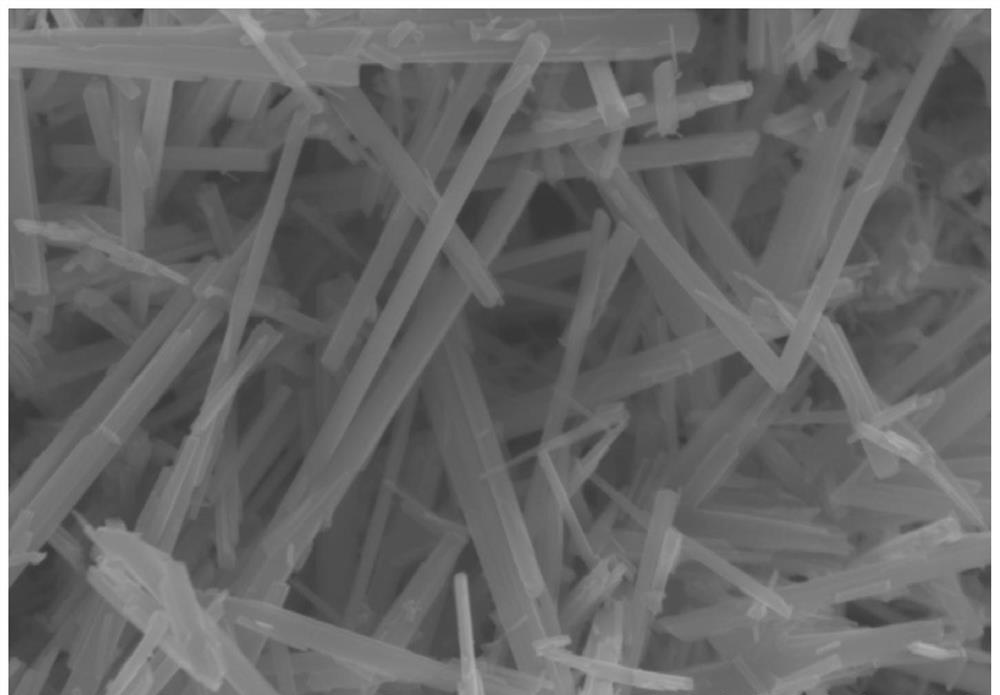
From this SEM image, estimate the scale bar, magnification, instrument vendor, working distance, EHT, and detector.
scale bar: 1000 nm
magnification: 45 K X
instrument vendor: Hitachi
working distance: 9.6 mm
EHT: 5 kV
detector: SE(UL)